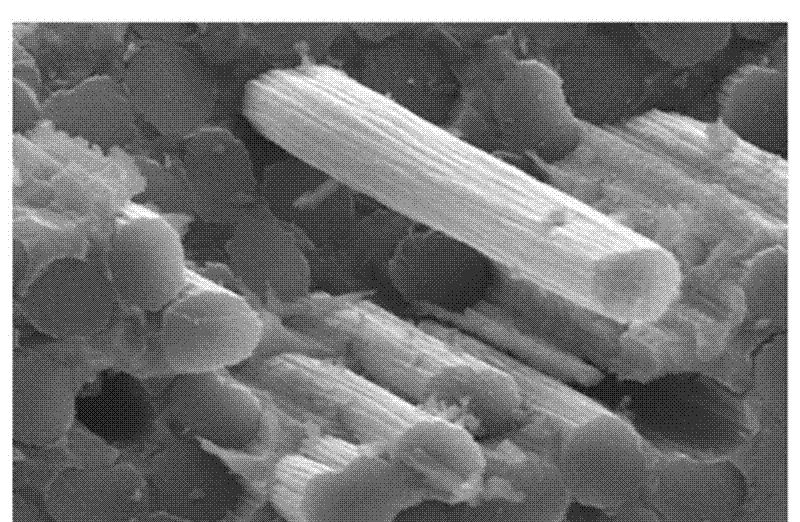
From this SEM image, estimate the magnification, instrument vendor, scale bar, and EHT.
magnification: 2 K X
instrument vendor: JEOL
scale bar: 10000 nm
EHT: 20 kV